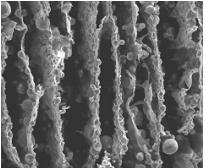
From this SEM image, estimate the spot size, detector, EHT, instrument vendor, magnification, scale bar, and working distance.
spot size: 4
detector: ETD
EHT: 20 kV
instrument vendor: FEI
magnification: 0.2 K X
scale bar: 400000 nm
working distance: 12.5 mm